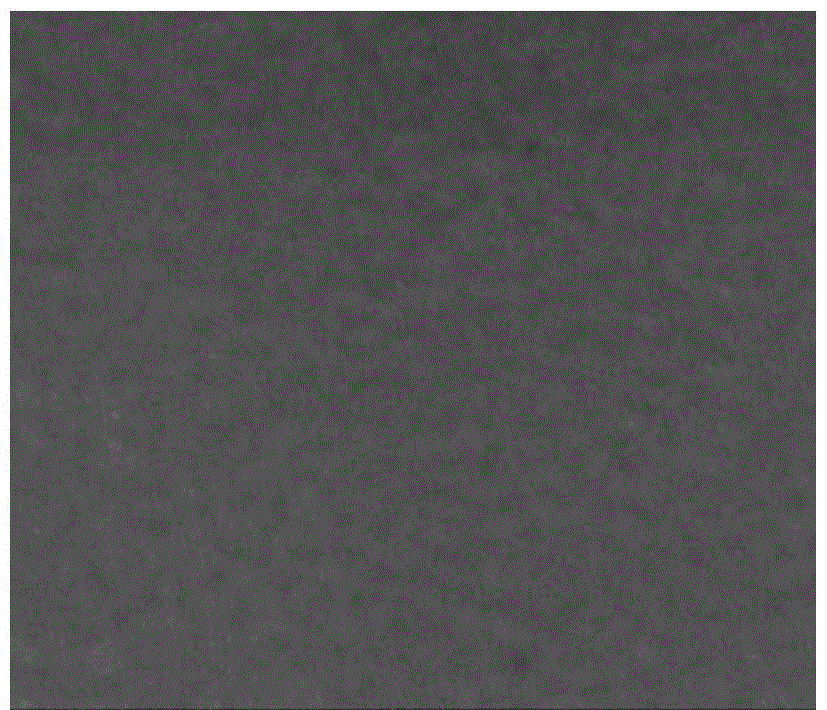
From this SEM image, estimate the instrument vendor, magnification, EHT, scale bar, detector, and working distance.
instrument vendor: FEI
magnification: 160 K X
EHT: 20 kV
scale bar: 500 nm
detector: SE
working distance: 9.8 mm